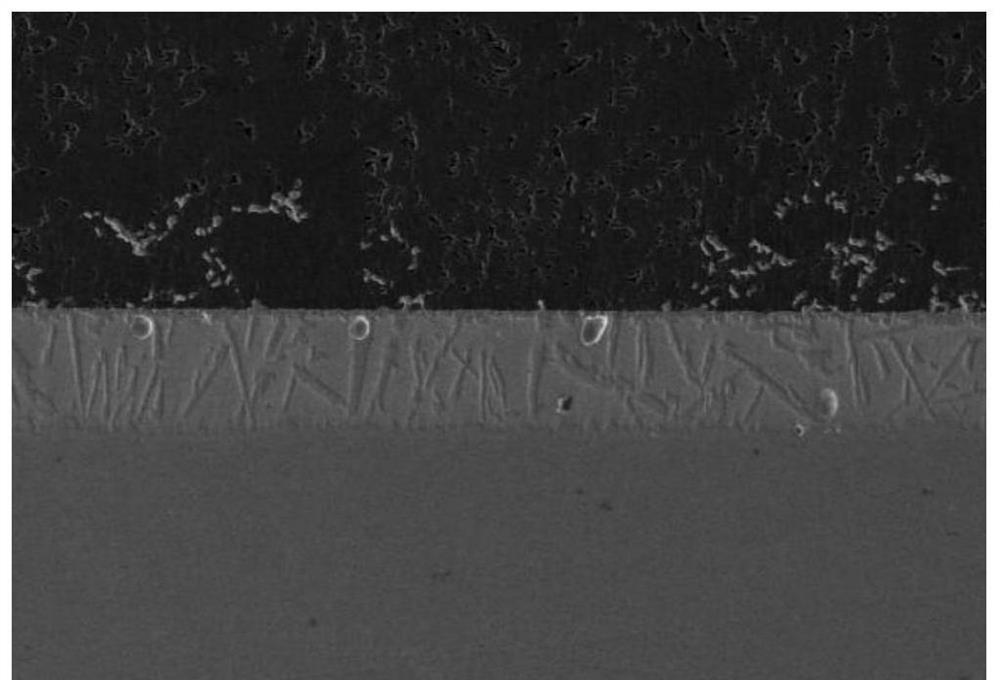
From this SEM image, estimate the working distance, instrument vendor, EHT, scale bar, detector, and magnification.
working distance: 13.8 mm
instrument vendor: Hitachi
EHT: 15 kV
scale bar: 500000 nm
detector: LM(L)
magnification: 0.1 K X